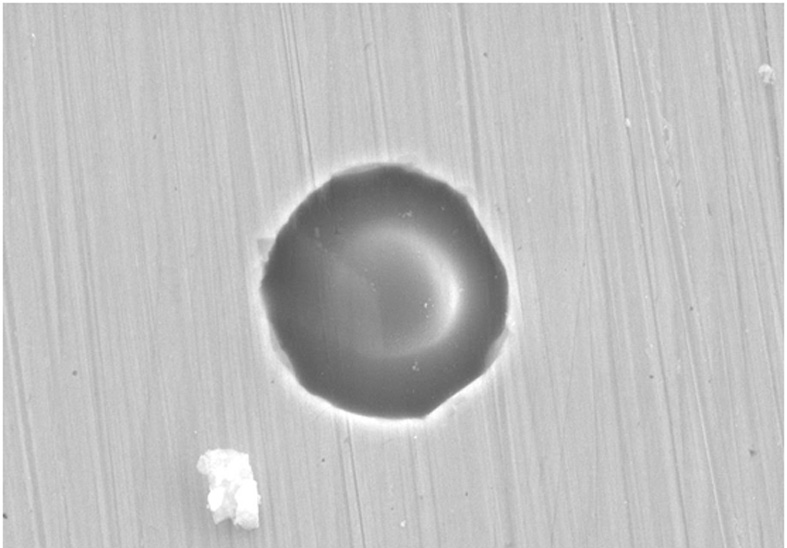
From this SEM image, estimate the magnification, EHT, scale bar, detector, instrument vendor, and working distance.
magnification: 6.5 K X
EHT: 15 kV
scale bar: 2000 nm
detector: SE2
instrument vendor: Zeiss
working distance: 9.1 mm